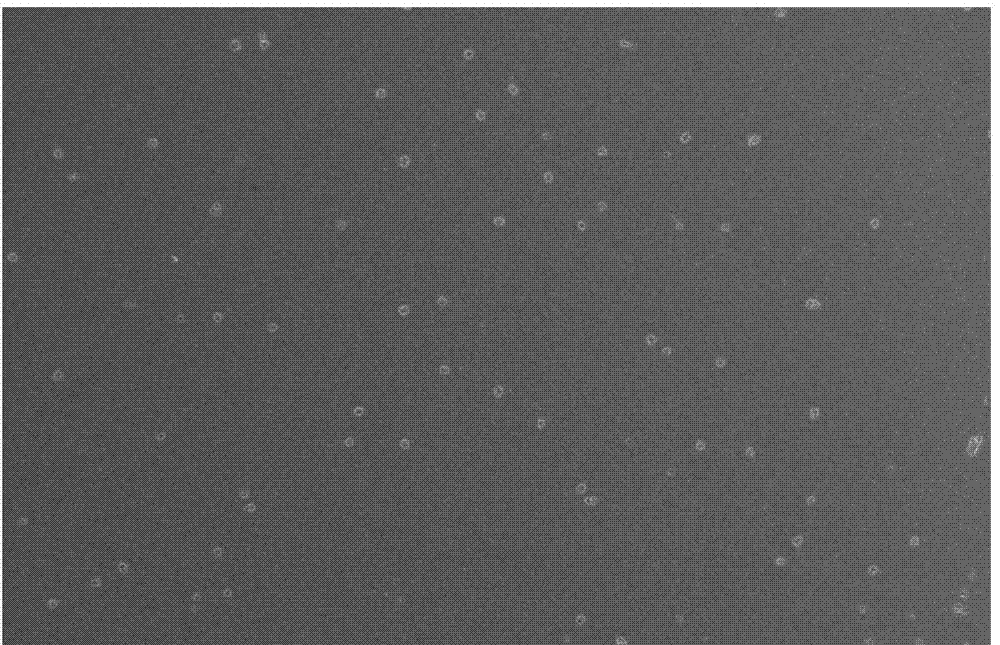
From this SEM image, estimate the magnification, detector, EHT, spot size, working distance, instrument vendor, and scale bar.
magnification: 5 K X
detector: TLD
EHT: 5 kV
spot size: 4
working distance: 5 mm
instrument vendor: FEI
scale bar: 10000 nm